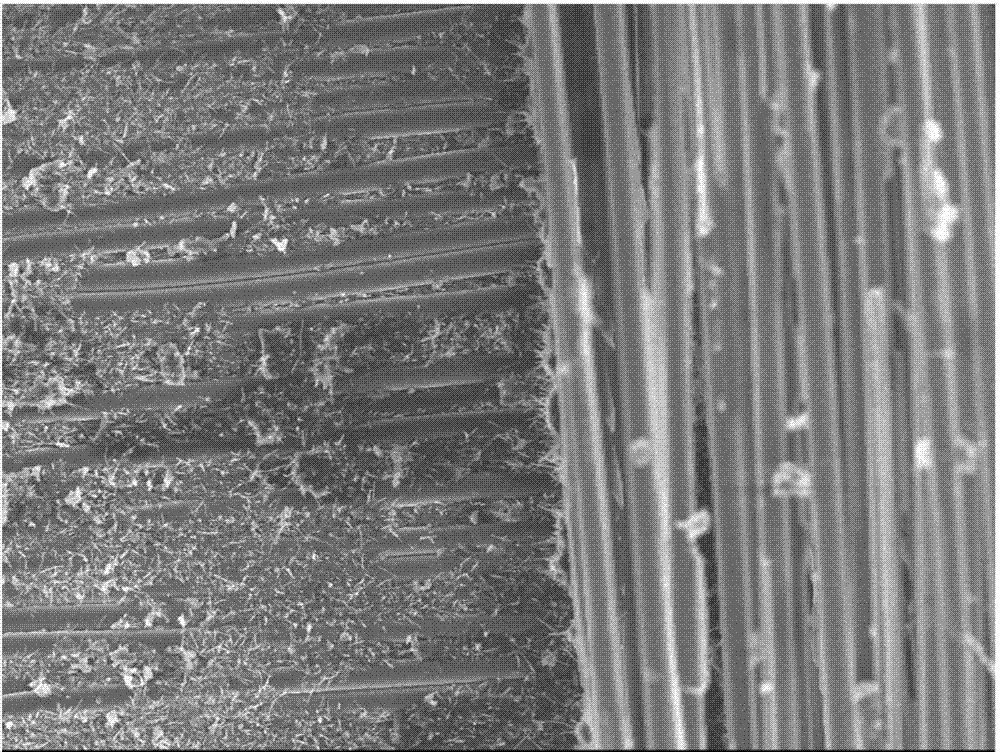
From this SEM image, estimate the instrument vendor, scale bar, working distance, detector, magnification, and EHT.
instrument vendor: JEOL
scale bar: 10000 nm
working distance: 8.7 mm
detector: SEI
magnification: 0.5 K X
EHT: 10 kV